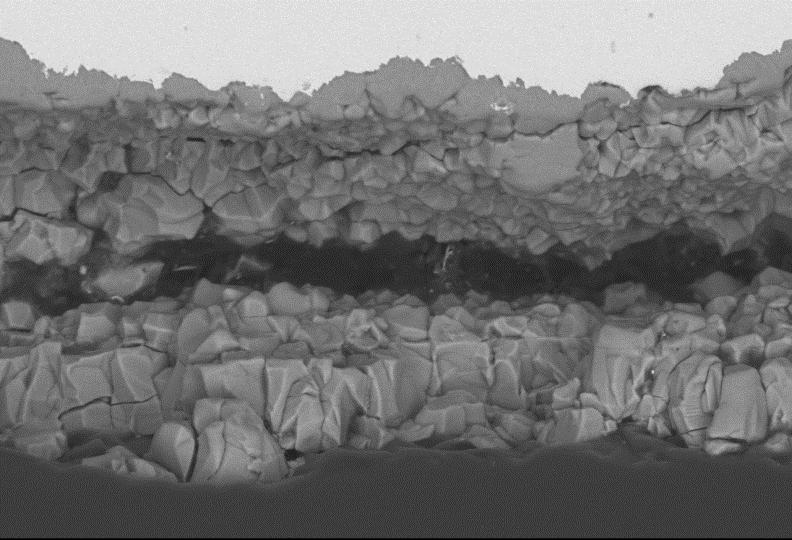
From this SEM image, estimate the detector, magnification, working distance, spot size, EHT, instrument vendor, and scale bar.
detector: BEC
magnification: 0.5 K X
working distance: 10 mm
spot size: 40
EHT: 20 kV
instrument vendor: JEOL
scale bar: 50000 nm